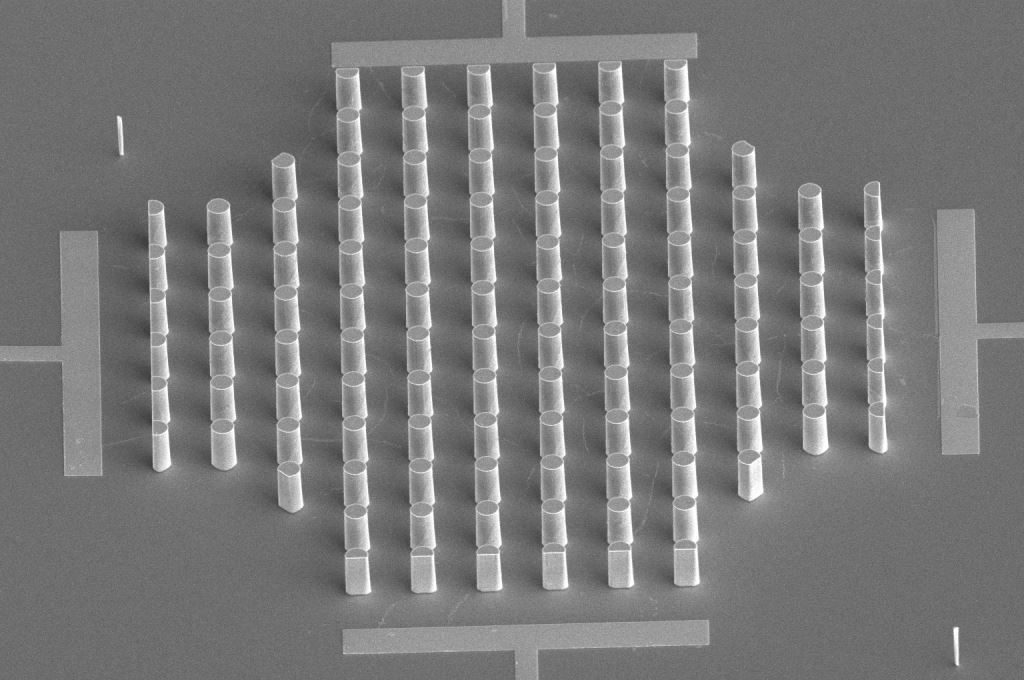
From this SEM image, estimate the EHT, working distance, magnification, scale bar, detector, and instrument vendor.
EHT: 5 kV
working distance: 20.4 mm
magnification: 1.5 K X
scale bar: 50000 nm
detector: ETD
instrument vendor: FEI